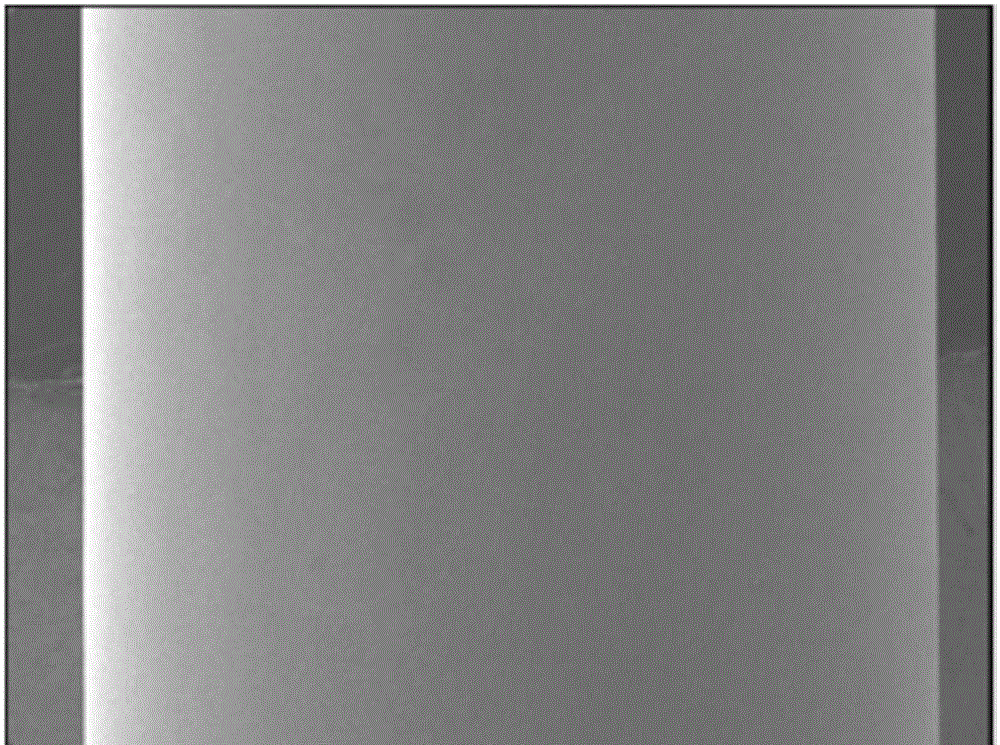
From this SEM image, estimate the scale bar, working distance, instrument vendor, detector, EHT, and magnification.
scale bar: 100000 nm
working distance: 8.1 mm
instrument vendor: JEOL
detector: LEI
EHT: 5 kV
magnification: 0.035 K X